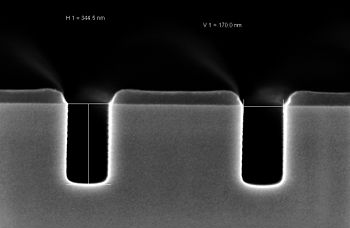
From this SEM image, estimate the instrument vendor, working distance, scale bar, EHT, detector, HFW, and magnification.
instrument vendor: Zeiss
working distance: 3.3 mm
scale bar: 100 nm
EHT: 2 kV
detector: InLens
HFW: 1.501 µm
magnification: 200 K X